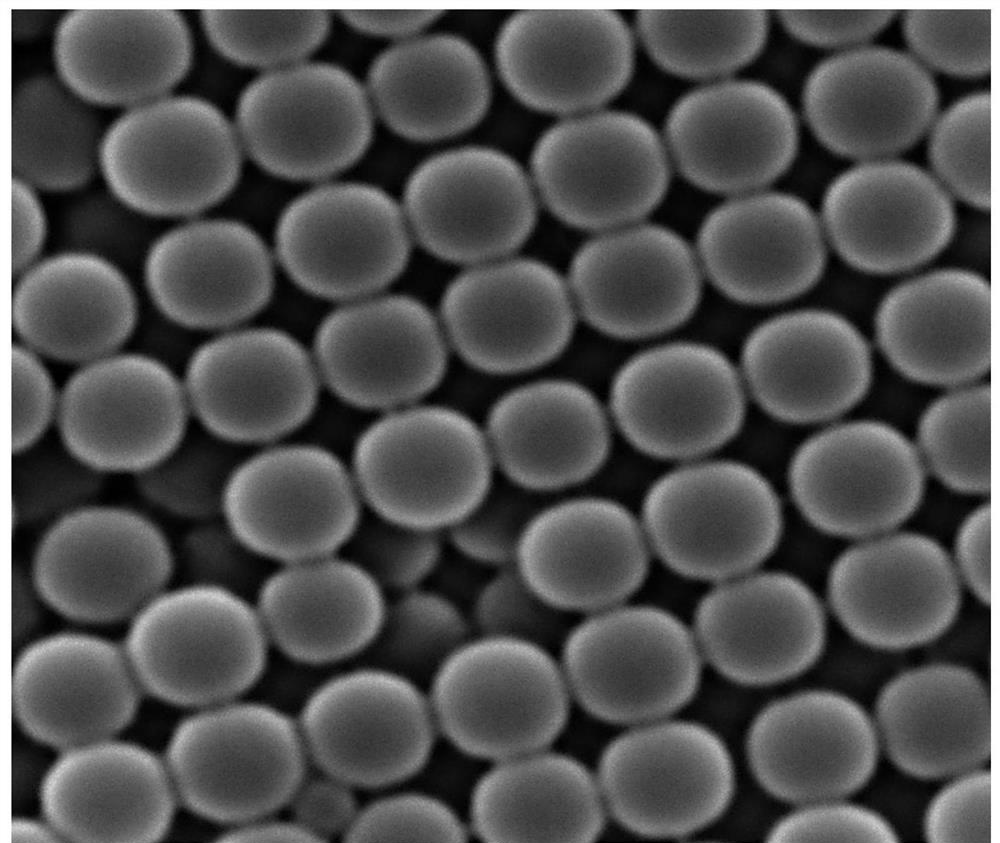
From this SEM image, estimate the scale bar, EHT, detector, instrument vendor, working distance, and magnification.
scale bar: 1000 nm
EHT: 30 kV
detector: SE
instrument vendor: Tescan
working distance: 15.08 mm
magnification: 35.1 K X